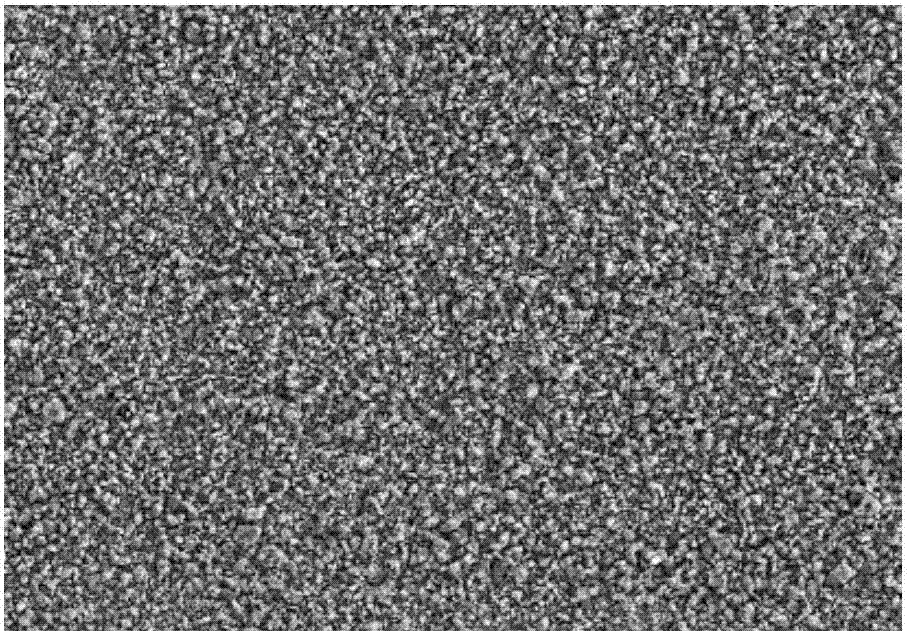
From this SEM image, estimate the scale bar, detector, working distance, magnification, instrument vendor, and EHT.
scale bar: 50000 nm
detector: SE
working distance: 7.8 mm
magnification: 1 K X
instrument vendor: Hitachi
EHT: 15 kV